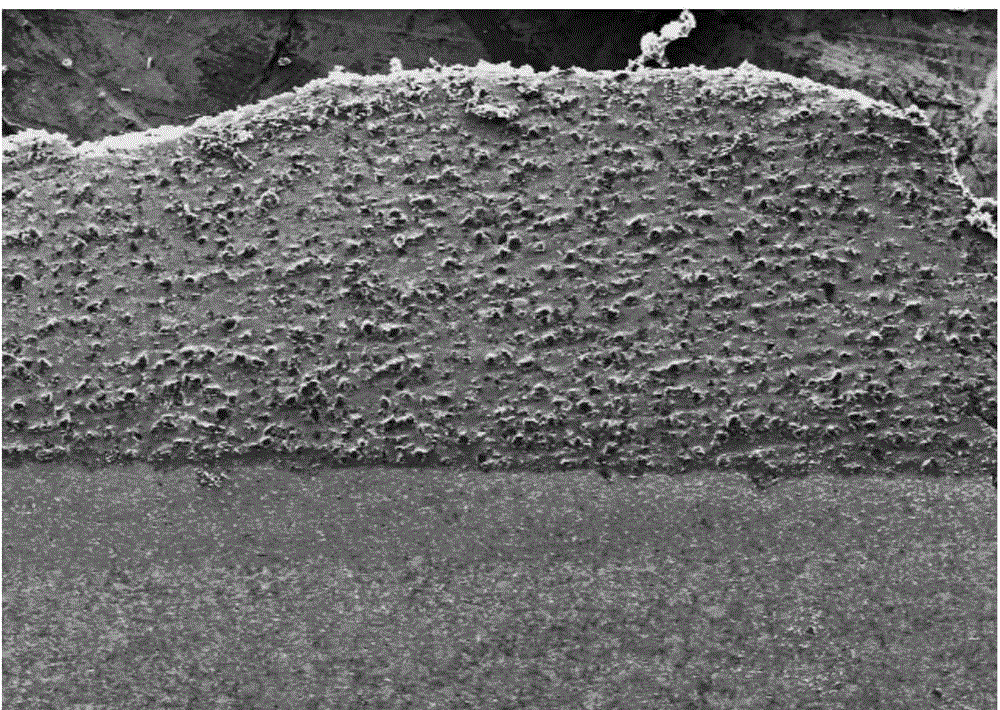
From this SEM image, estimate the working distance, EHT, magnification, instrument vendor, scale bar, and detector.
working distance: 13.7 mm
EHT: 15 kV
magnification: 0.05 K X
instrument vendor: Hitachi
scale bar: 1000000 nm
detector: SE(M)